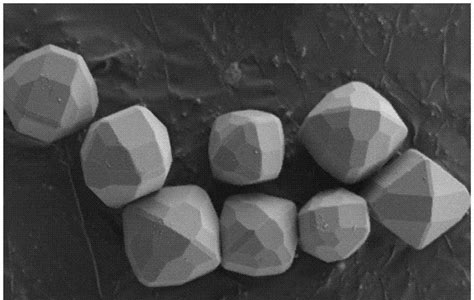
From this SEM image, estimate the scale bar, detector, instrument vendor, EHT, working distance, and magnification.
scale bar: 2000 nm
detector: SE2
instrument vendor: Zeiss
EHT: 1 kV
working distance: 6.2 mm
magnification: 9 K X